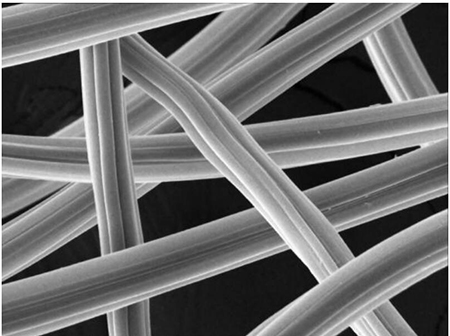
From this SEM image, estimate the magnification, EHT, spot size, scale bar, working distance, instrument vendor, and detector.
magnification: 2 K X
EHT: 25 kV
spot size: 5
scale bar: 6000 nm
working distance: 46.27 mm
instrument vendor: other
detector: SEI